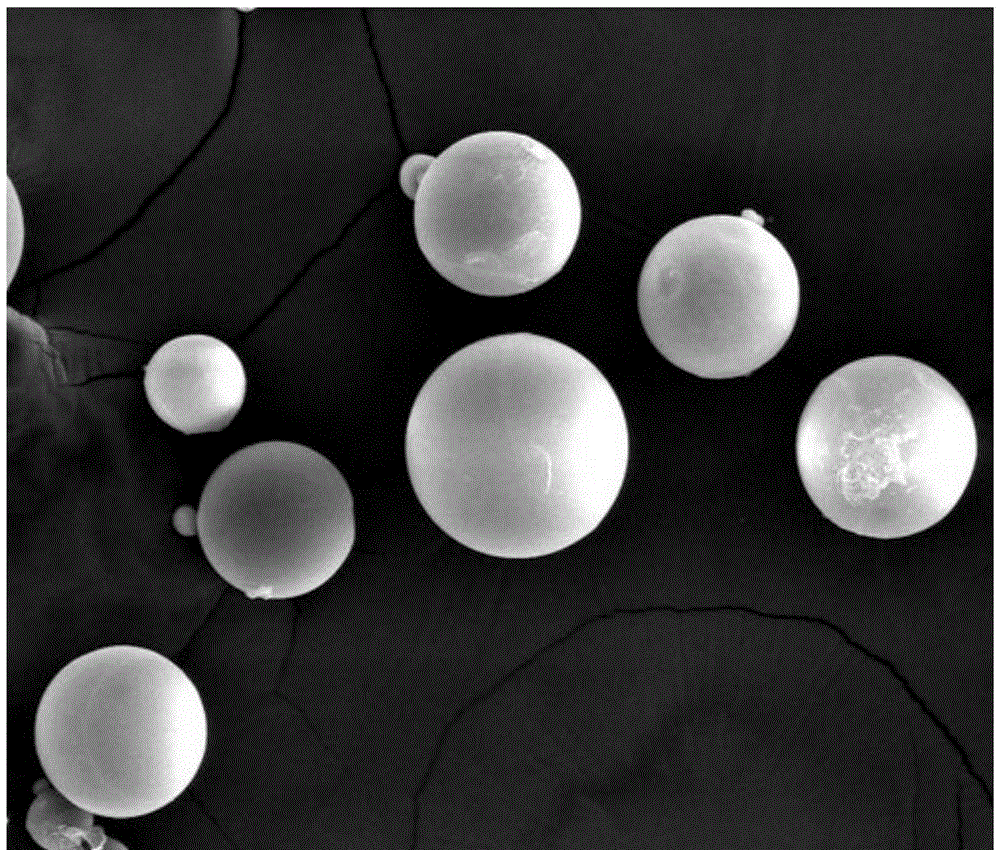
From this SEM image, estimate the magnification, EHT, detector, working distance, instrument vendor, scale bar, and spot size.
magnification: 0.8 K X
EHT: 25 kV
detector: SE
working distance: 18.7 mm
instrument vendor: FEI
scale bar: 100000 nm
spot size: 4